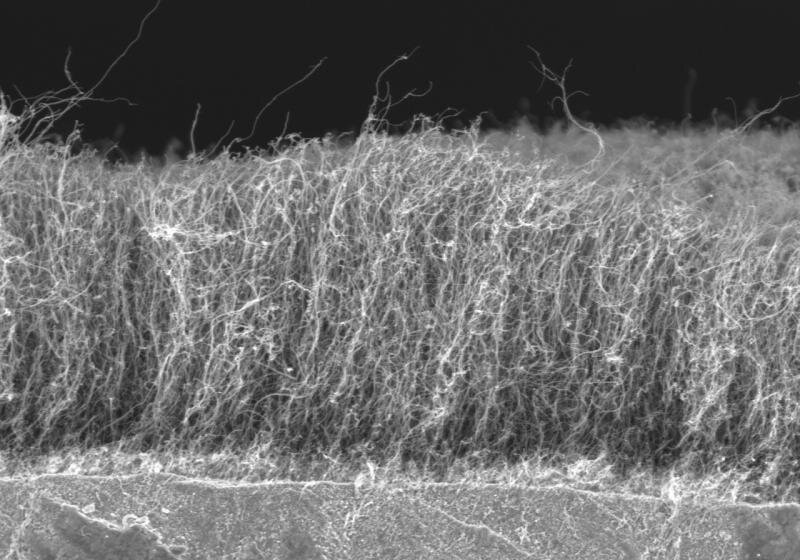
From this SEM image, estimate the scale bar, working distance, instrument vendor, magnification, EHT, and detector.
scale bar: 10000 nm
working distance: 10.4 mm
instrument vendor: Hitachi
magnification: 3 K X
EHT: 5 kV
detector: SE(U)